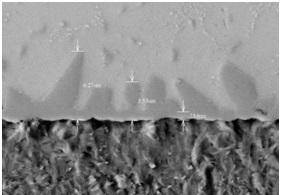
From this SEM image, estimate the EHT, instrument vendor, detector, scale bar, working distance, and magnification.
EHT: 15 kV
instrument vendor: Hitachi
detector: BSE3D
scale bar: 10000 nm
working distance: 7.9 mm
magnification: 3 K X